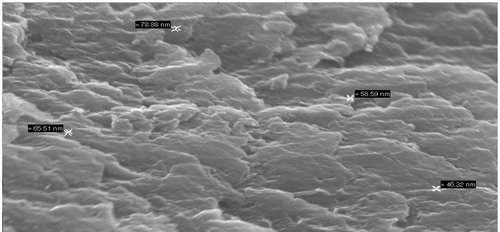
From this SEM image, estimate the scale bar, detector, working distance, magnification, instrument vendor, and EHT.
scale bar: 1000 nm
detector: SE1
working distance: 8.5 mm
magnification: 20 K X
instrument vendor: Zeiss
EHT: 20 kV